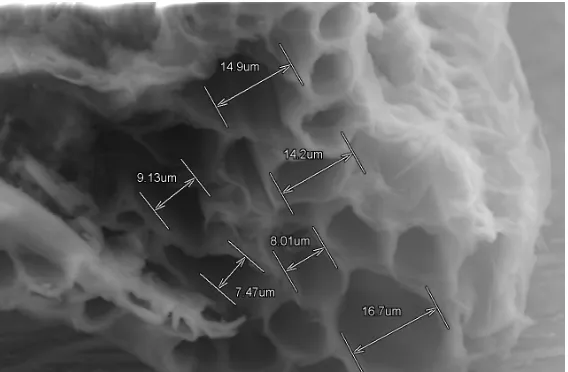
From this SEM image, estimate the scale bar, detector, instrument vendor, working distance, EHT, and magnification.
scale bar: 40000 nm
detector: SE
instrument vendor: Hitachi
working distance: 33.2 mm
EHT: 30 kV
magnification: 1.3 K X